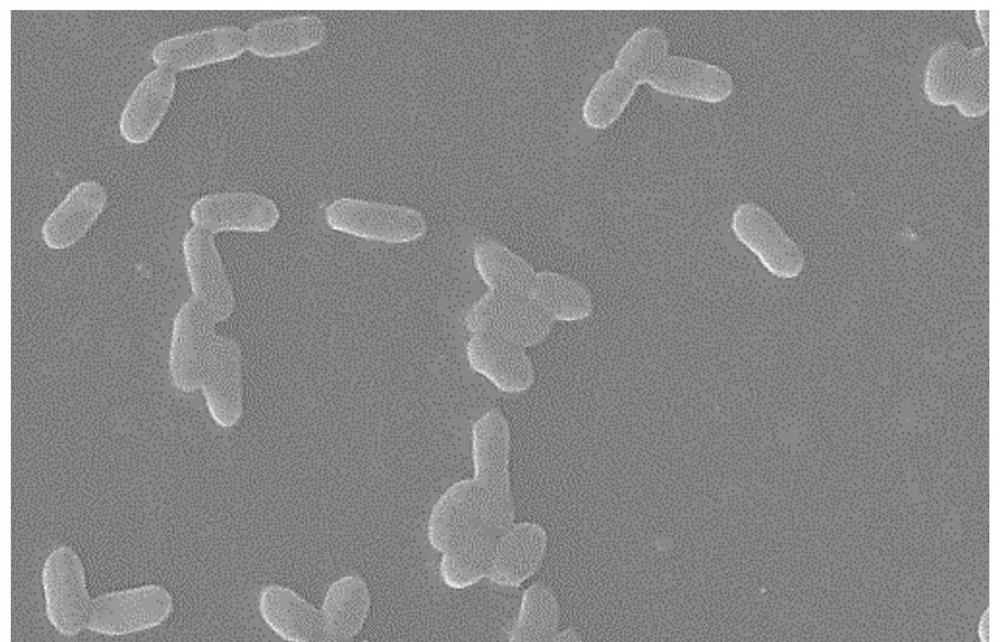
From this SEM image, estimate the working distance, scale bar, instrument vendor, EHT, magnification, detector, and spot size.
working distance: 10 mm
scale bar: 2000 nm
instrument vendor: FEI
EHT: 10 kV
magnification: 8 K X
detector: SE2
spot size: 3